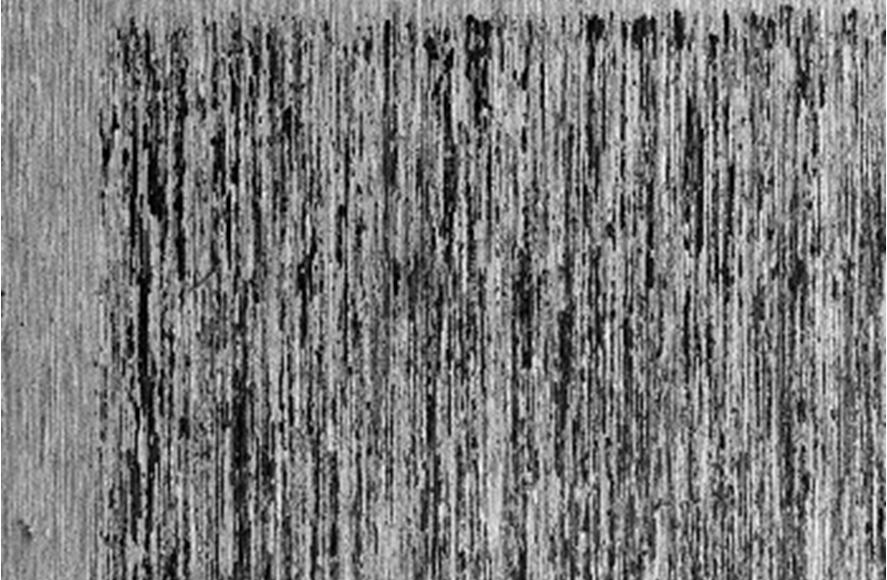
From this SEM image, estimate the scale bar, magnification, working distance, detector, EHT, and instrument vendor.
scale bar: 1000000 nm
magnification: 0.025 K X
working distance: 16 mm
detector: LEI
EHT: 5 kV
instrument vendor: JEOL